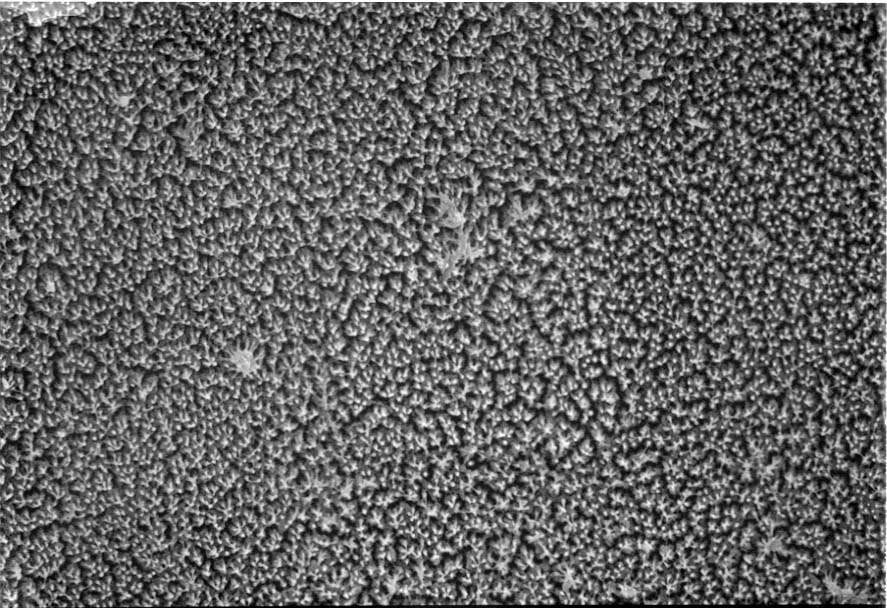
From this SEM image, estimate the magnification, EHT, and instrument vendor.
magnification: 2 K X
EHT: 20 kV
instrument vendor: JEOL